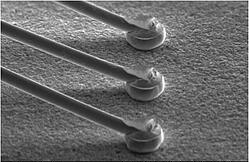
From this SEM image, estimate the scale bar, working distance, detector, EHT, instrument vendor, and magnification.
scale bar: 200000 nm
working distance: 27.5 mm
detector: SE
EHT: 25 kV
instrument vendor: JEOL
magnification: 0.18 K X